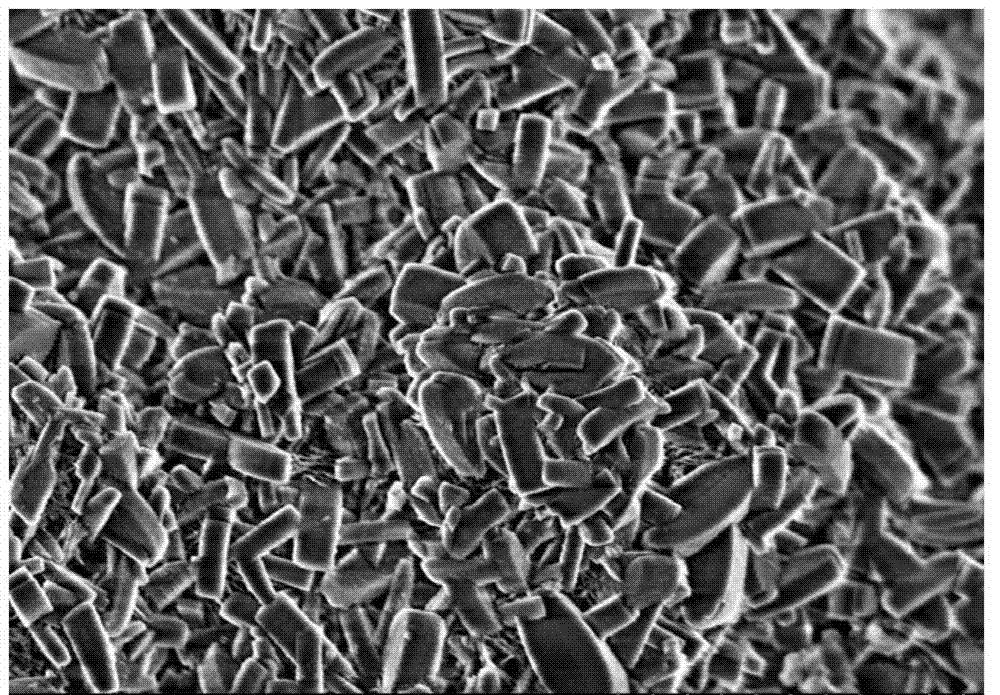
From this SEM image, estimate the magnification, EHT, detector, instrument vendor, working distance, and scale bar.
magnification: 9 K X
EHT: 3 kV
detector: SE(M)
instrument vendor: Hitachi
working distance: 3.3 mm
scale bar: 5000 nm